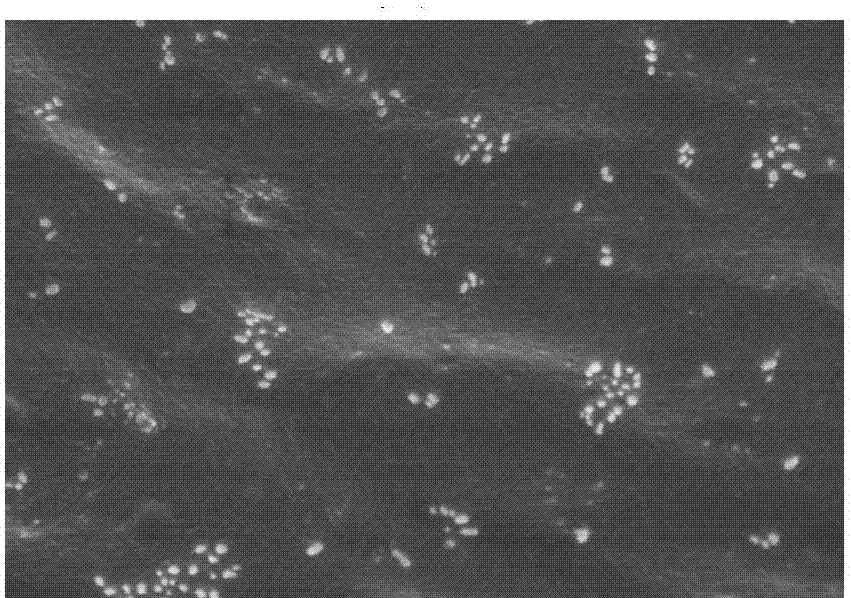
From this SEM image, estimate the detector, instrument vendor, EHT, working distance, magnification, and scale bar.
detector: SE(U)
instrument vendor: Hitachi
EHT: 5 kV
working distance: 6.3 mm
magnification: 20 K X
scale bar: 2000 nm